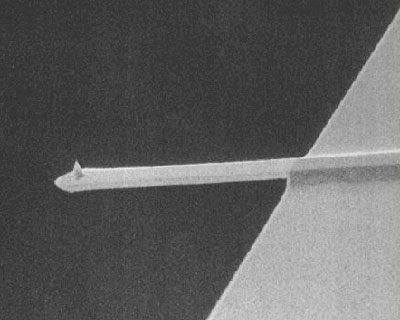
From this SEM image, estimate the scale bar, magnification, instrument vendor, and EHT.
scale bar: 100000 nm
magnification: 0.3 K X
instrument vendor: JEOL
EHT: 25 kV